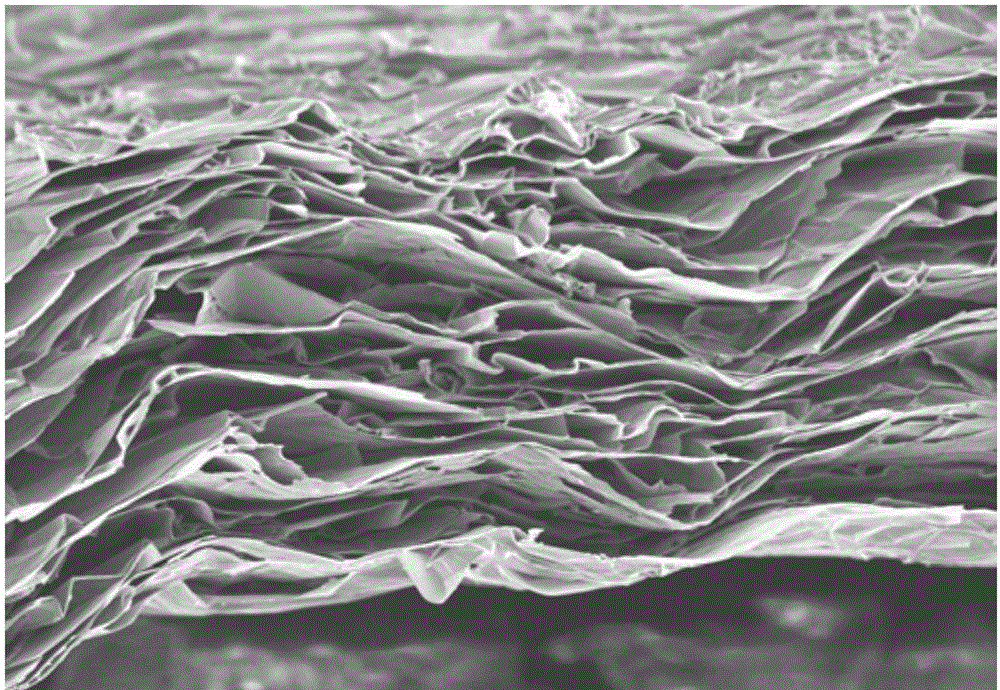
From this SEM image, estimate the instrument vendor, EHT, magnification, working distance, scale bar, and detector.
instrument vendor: Hitachi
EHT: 3 kV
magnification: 2 K X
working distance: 13 mm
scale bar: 20000 nm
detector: SE(M)